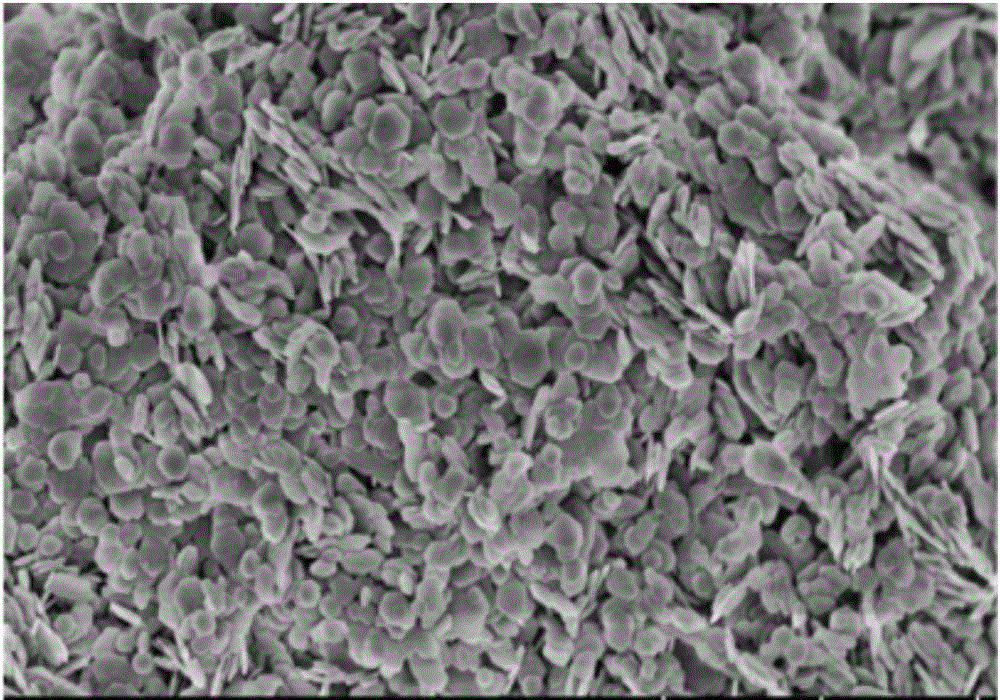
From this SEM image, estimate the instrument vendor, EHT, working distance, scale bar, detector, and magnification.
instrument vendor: Hitachi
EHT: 2 kV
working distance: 5.2 mm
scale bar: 2000 nm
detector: SE(U)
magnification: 20 K X